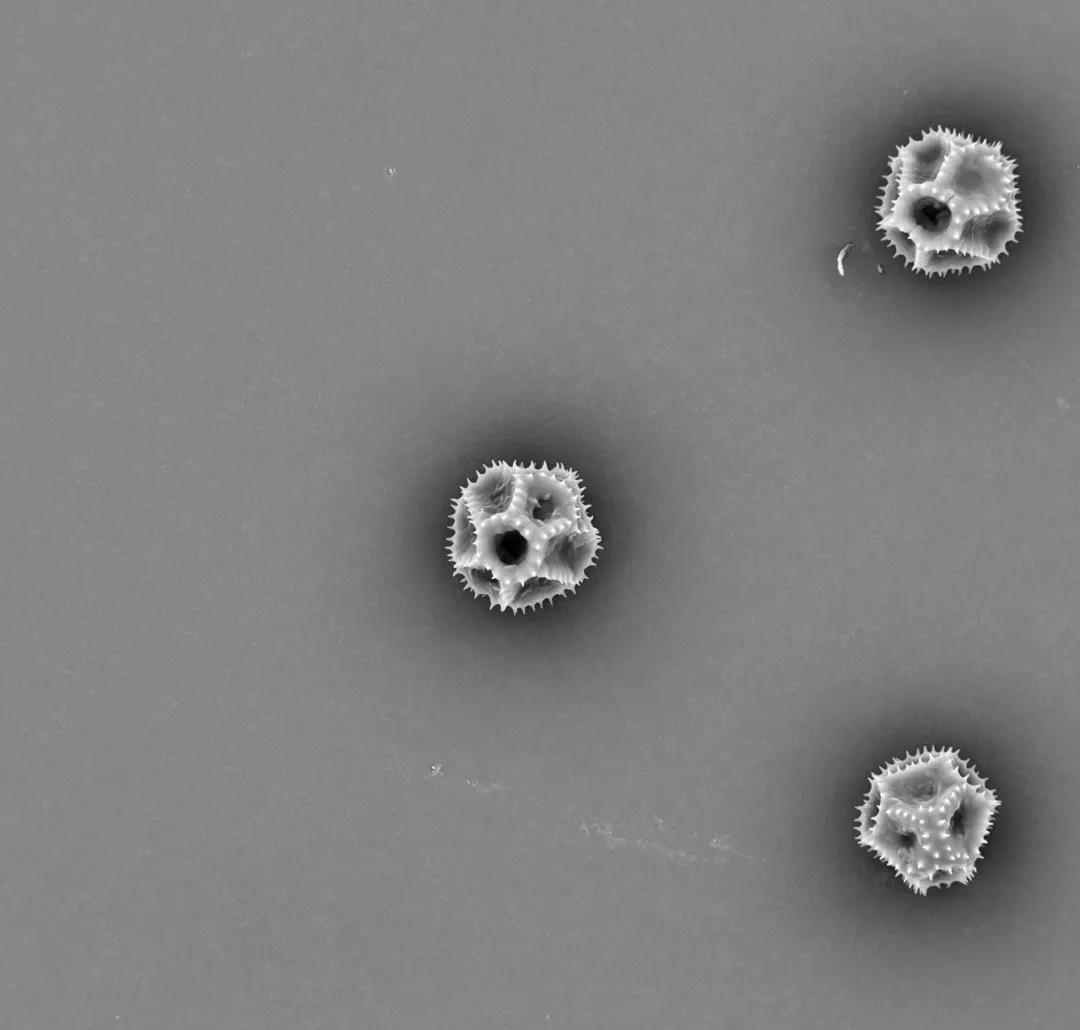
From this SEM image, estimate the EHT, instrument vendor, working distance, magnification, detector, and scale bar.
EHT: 15 kV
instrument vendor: other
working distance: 5.5 mm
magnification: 1 K X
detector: BSE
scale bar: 50000 nm